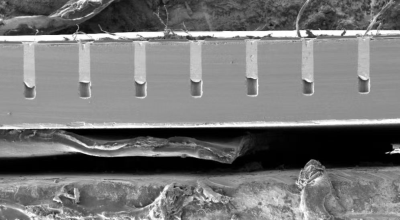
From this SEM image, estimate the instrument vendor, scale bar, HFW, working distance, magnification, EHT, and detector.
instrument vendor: Tescan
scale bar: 500000 nm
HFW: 2540 µm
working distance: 7.87 mm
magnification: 0.1 K X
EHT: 5 kV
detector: SE